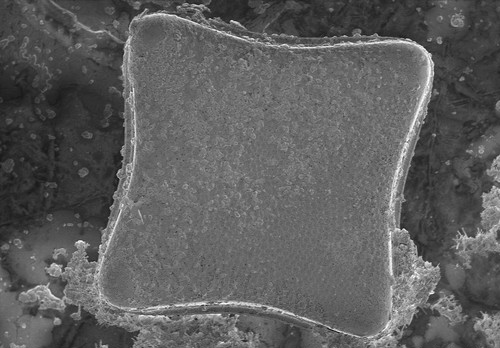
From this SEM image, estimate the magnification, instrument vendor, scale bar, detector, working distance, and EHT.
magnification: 0.45 K X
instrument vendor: Hitachi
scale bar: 100000 nm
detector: SE(M)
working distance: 9.4 mm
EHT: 1 kV